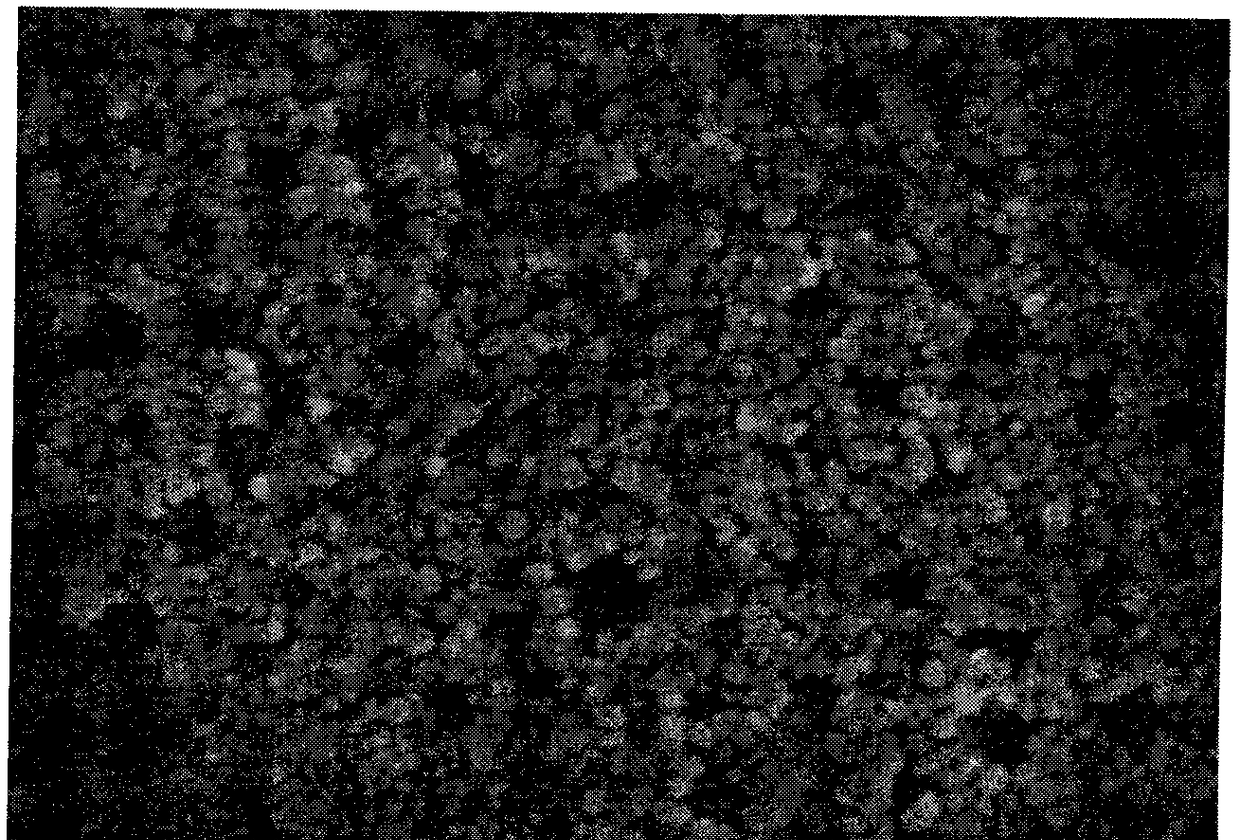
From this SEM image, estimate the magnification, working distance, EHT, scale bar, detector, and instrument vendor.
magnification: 100 K X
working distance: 7.8 mm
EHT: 2 kV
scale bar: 500 nm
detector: SE(U)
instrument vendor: Hitachi